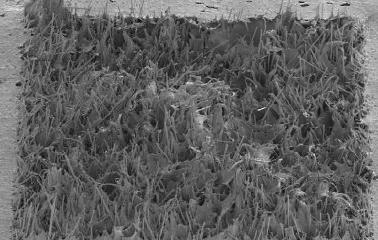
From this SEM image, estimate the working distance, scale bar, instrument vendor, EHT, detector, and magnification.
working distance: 22 mm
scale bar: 1000000 nm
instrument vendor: Hitachi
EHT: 5 kV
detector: SE(M)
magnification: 0.03 K X